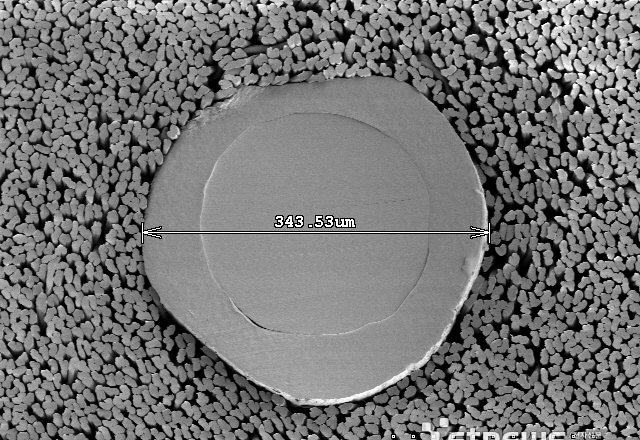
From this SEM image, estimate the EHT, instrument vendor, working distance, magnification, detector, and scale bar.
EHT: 1 kV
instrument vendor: JEOL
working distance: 14.5 mm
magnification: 0.2 K X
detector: SE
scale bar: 200000 nm